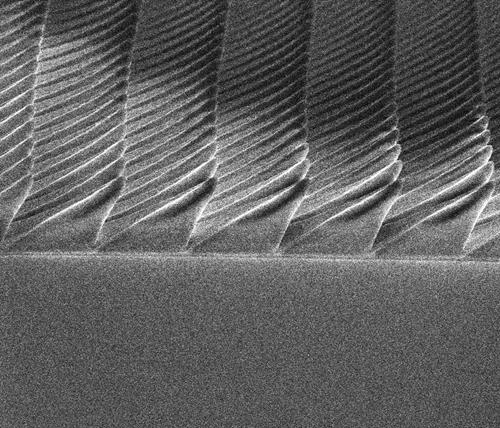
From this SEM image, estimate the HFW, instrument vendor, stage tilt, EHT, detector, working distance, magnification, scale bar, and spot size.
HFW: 27.1 µm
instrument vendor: FEI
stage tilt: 45°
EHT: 5 kV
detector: ETD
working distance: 4.8 mm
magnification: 11 K X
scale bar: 5000 nm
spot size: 2